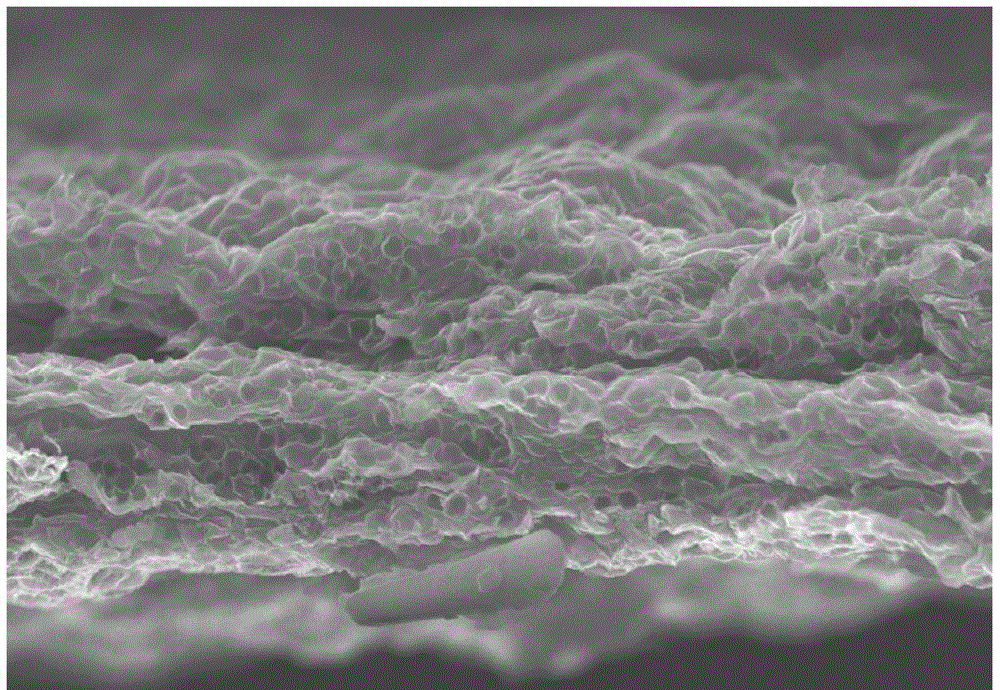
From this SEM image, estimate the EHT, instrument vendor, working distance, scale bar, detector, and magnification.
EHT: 10 kV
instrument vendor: Hitachi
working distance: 10 mm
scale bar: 5000 nm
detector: SE(U)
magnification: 9 K X